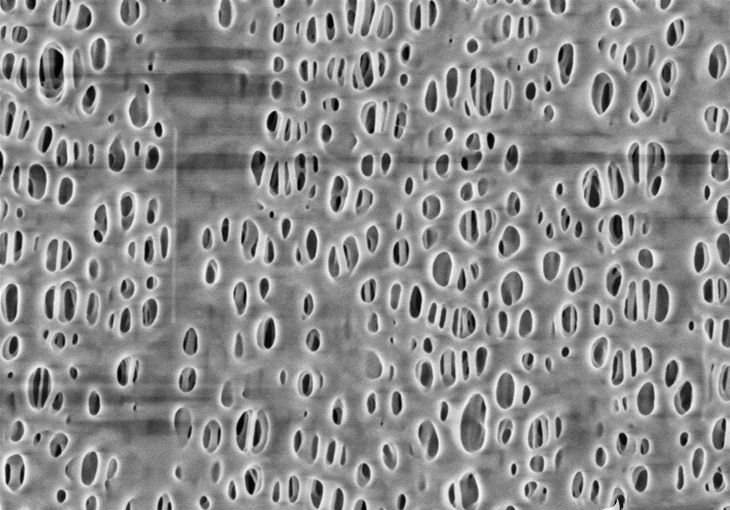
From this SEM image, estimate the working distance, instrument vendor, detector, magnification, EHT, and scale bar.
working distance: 1.5 mm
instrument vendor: Hitachi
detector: SE(U,LA0)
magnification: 35 K X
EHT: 1 kV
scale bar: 1000 nm